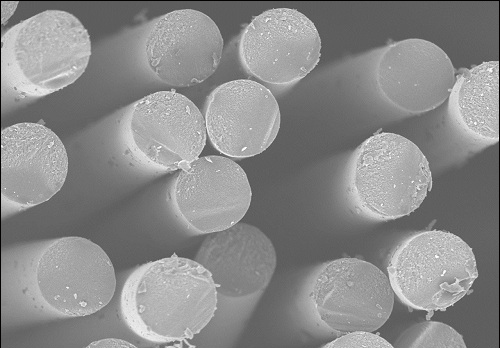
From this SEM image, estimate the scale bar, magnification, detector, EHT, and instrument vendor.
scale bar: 20000 nm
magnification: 2 K X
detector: SE(U)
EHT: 4 kV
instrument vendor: Hitachi